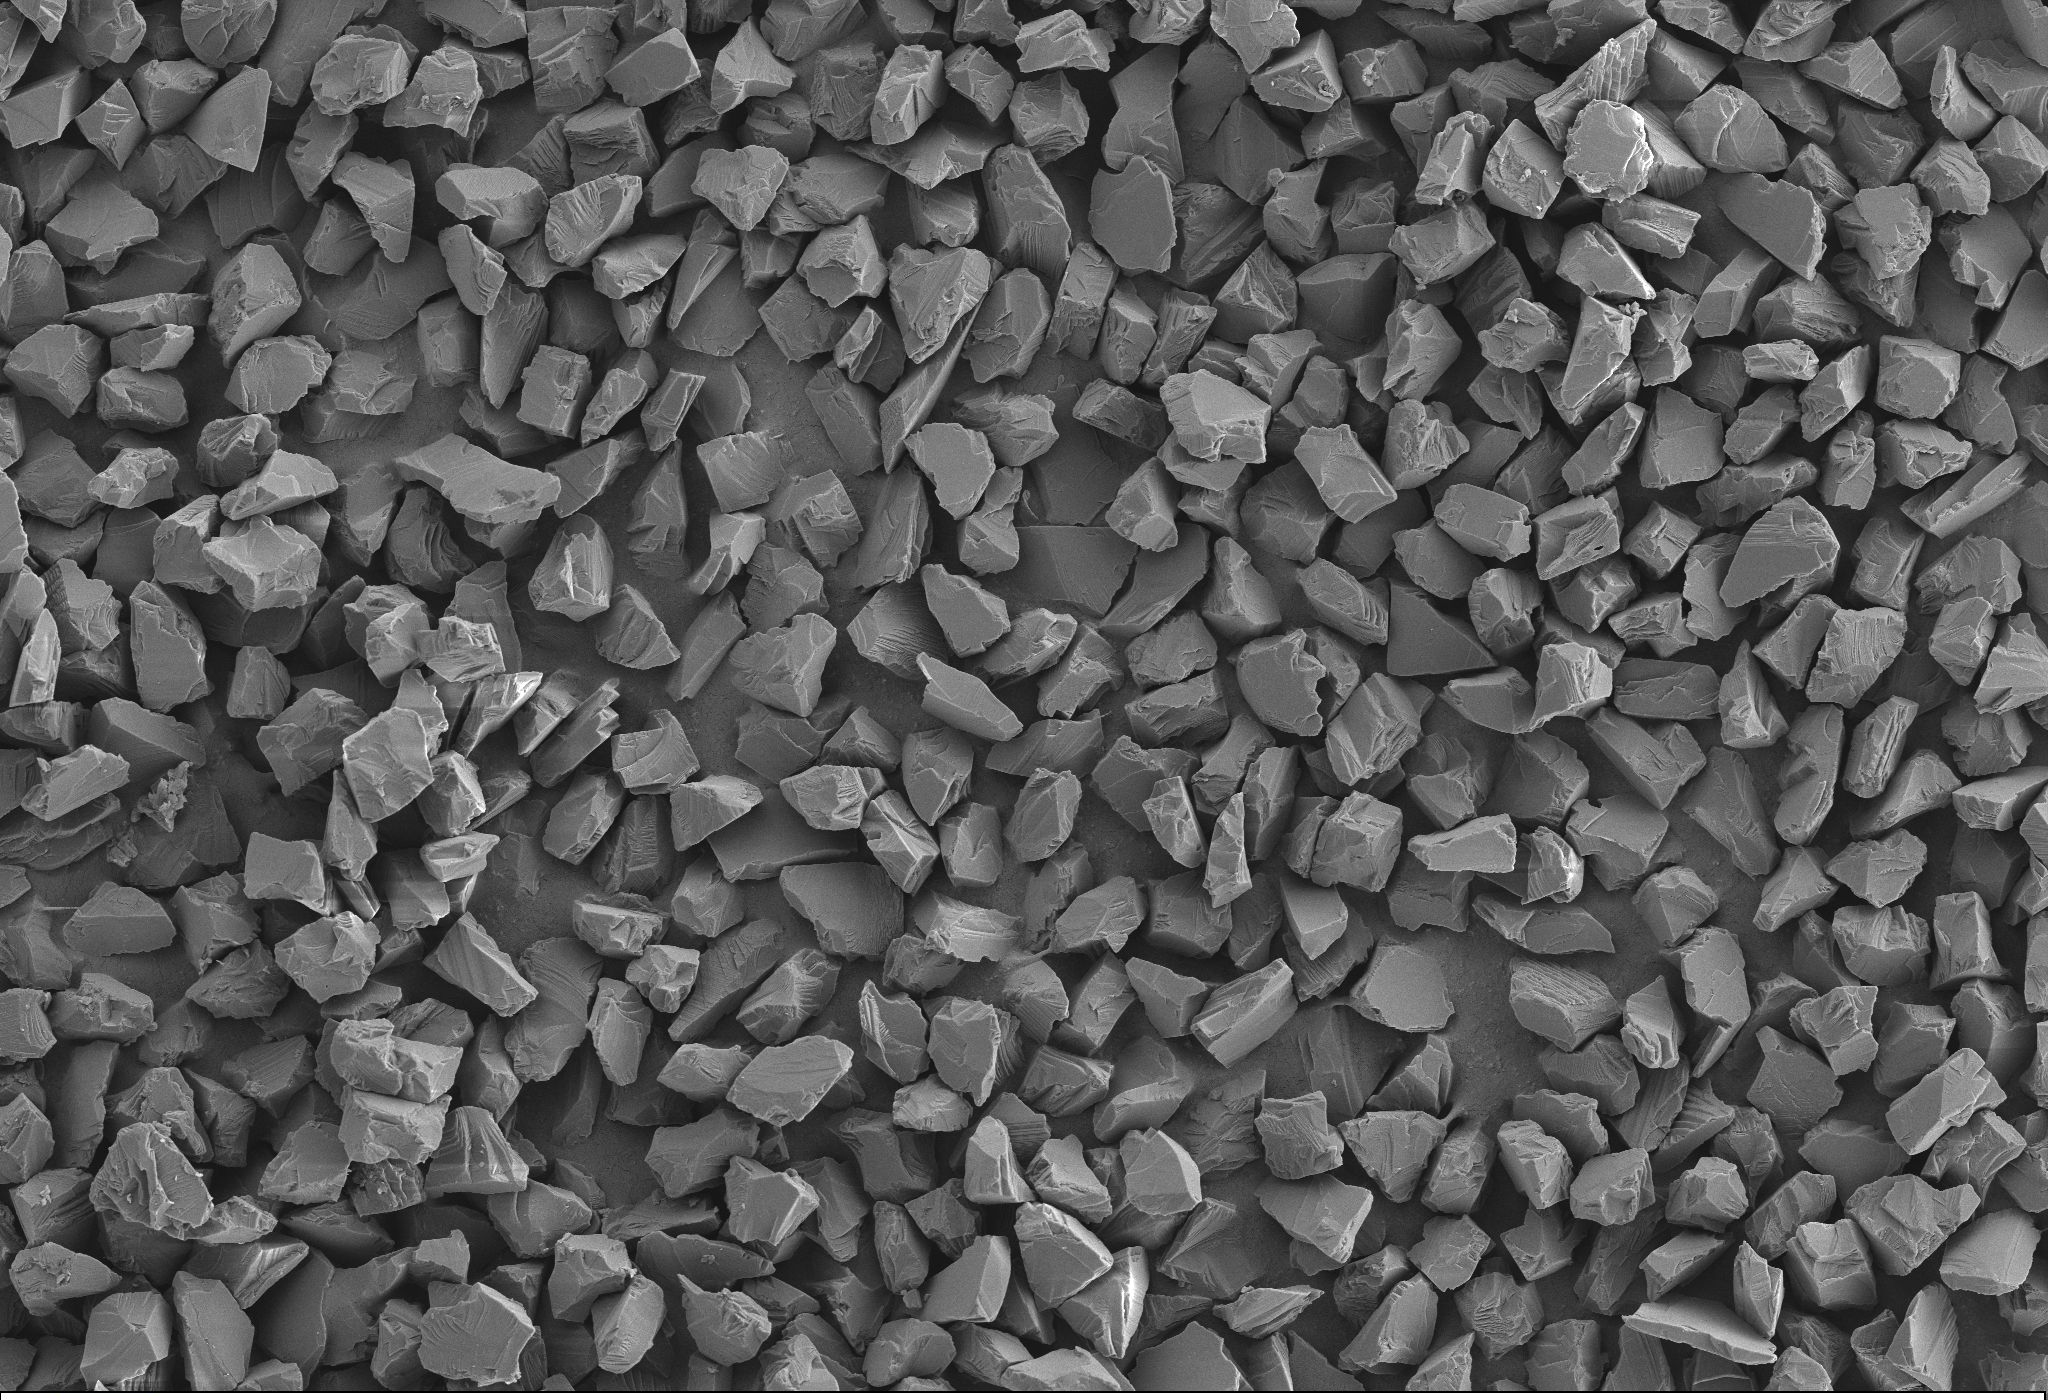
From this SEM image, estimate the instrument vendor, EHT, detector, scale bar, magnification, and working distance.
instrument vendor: Zeiss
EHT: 5 kV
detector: SE2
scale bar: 10000 nm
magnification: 2 K X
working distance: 7.2 mm